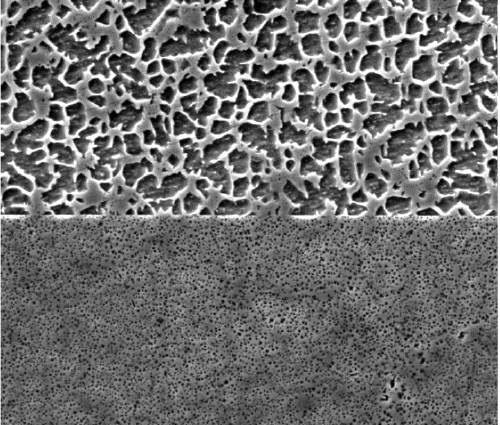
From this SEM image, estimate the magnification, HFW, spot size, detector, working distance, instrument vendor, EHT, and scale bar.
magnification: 5 K X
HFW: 54.08 µm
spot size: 4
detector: ETD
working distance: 9.1 mm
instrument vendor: FEI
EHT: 20 kV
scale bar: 10000 nm